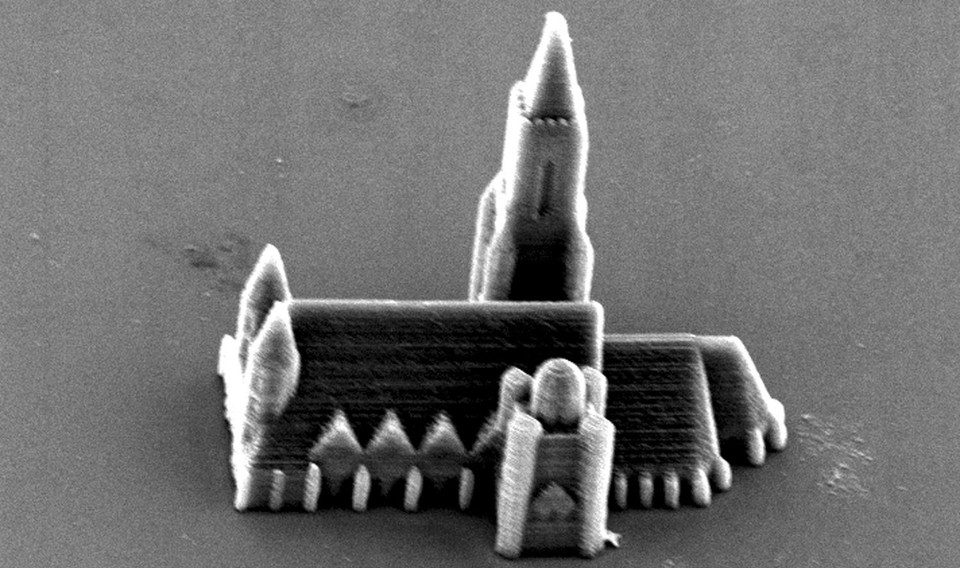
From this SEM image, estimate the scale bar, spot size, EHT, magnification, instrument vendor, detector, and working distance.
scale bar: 20000 nm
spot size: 4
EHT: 15 kV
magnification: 0.859 K X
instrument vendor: FEI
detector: SE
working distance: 27.5 mm